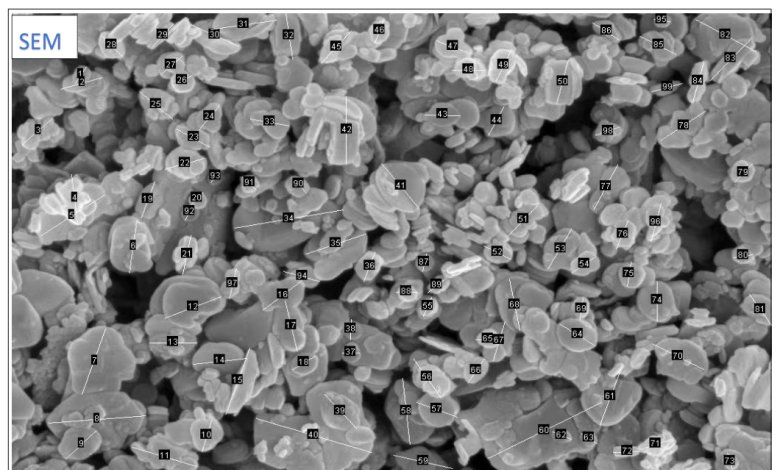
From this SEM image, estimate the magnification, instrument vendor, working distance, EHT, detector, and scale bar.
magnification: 30 K X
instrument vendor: Zeiss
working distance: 6.4 mm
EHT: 5 kV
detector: InLens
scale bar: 200 nm